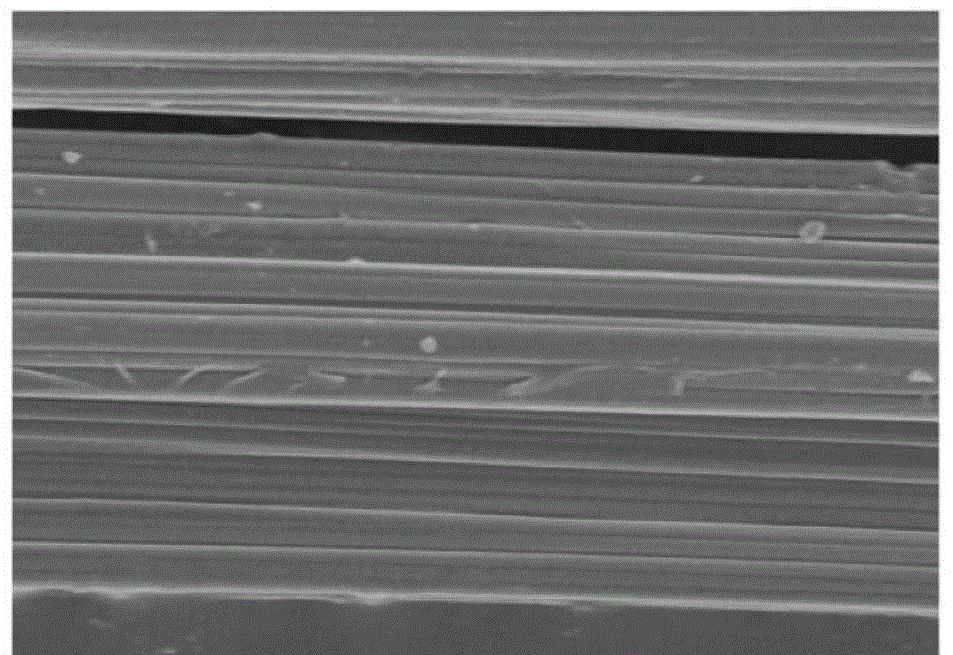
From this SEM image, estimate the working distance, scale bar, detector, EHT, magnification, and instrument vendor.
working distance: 8 mm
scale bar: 10000 nm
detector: SE(M)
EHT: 5 kV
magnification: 4 K X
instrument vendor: Hitachi